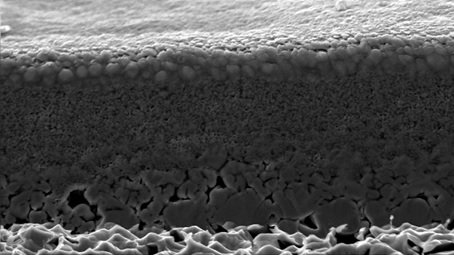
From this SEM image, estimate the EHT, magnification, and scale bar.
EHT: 30 kV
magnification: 1.62 K X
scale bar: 50000 nm